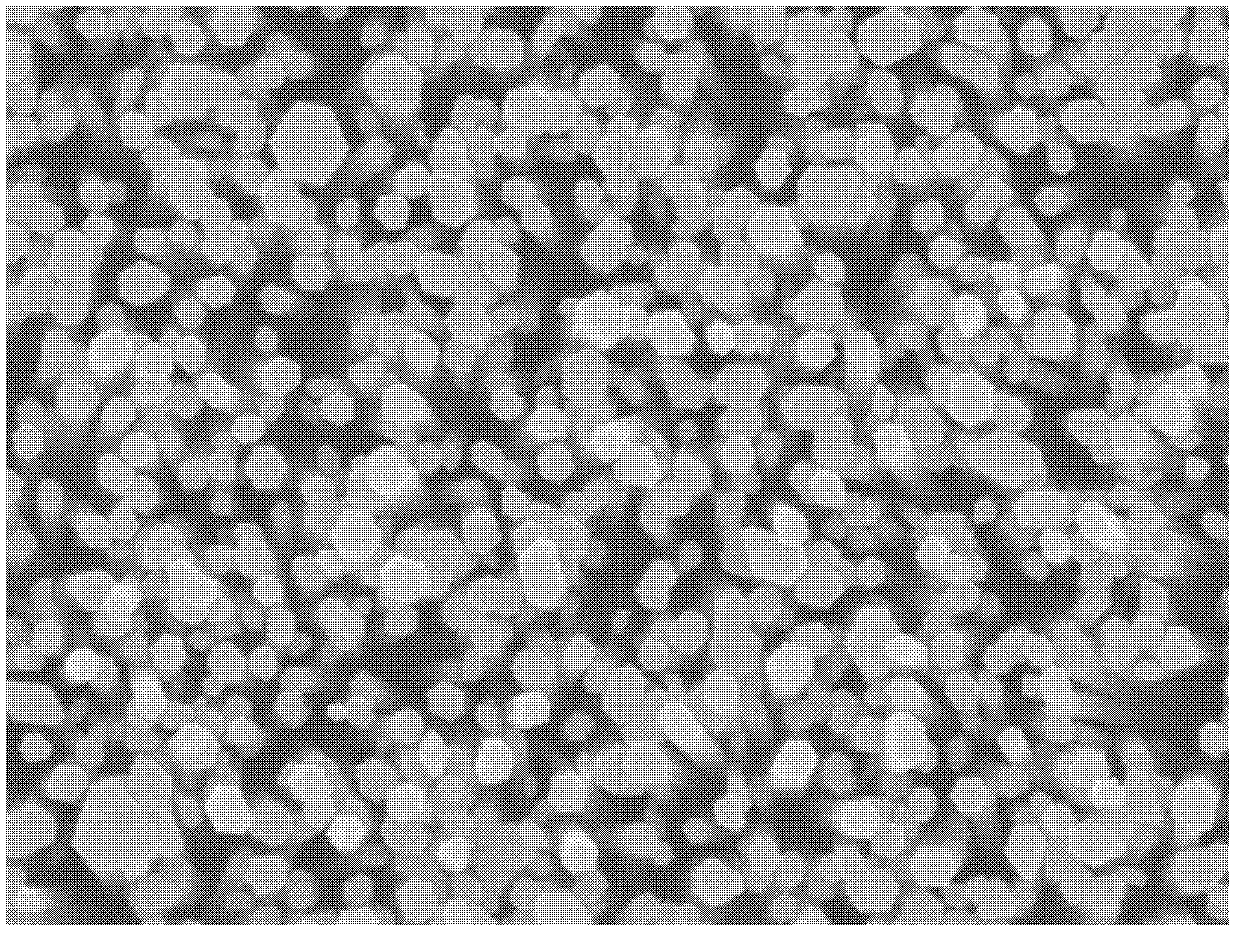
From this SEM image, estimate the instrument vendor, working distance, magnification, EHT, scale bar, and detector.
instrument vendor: JEOL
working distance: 6.8 mm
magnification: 30 K X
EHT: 10 kV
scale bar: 100 nm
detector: SEI